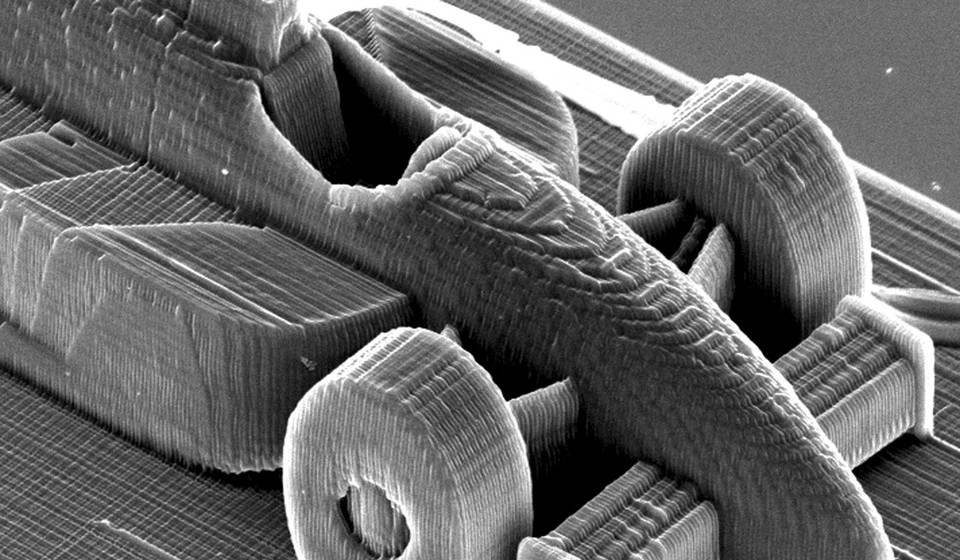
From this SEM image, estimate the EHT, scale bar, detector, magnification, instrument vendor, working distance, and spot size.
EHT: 20 kV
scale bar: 50000 nm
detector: SE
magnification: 0.656 K X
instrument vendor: FEI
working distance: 27.2 mm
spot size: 4